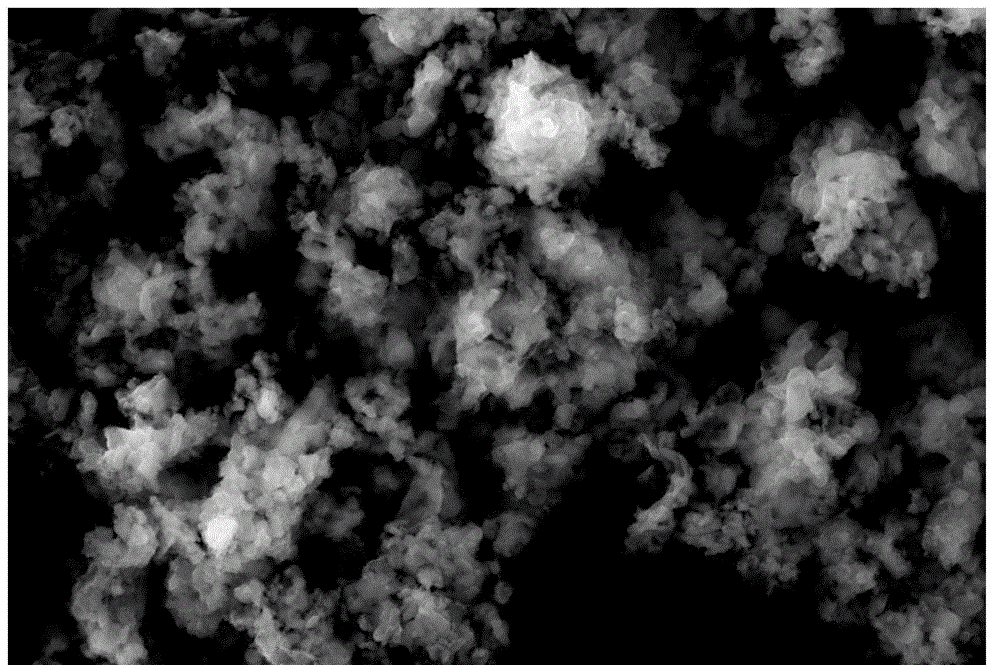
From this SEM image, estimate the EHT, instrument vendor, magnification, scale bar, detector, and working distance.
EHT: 30 kV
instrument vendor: JEOL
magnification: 5 K X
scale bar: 5000 nm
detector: SEI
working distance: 11 mm